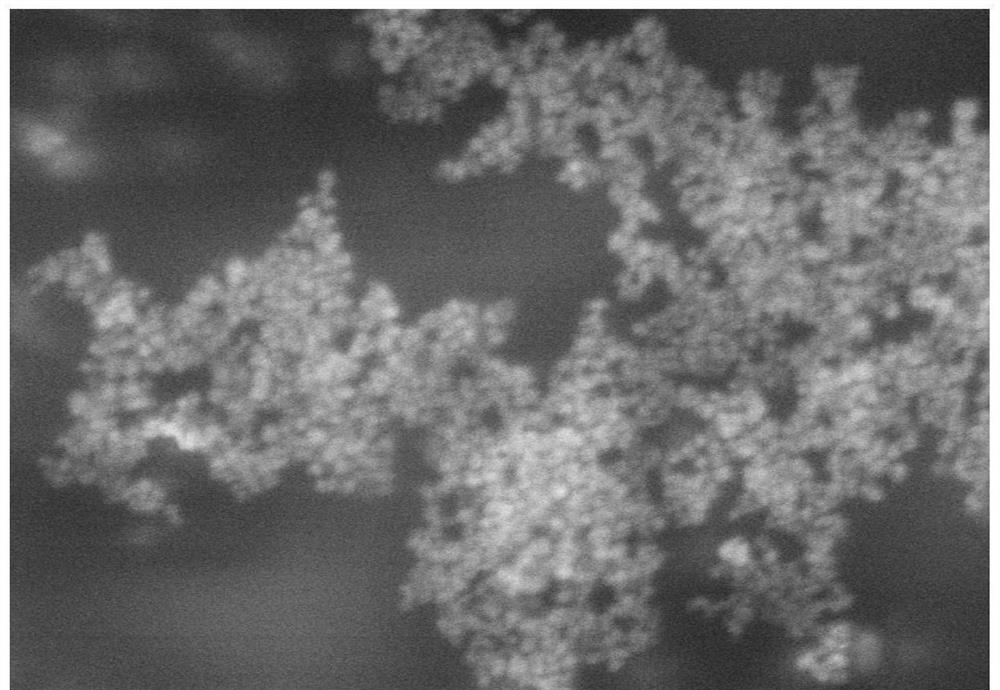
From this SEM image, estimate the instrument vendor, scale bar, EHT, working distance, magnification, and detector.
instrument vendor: Hitachi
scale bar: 1000 nm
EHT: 5 kV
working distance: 8.7 mm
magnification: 50 K X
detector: SE(TUL)